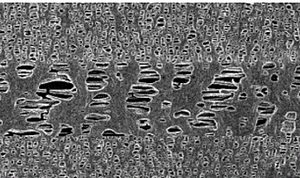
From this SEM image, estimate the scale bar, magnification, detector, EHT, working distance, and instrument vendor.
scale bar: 2000 nm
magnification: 20 K X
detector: SE(U)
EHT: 5 kV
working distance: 9.3 mm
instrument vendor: Hitachi